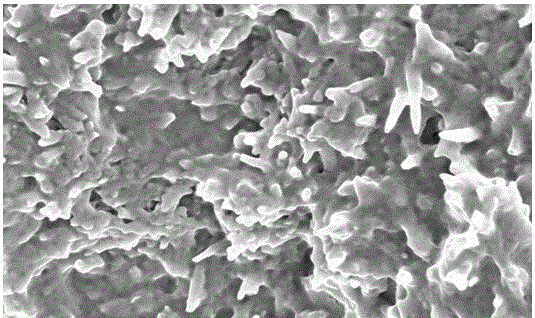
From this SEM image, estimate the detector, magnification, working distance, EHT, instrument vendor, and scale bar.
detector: SE(M)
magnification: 40 K X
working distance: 9.3 mm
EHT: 3 kV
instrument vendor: Hitachi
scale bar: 1000 nm